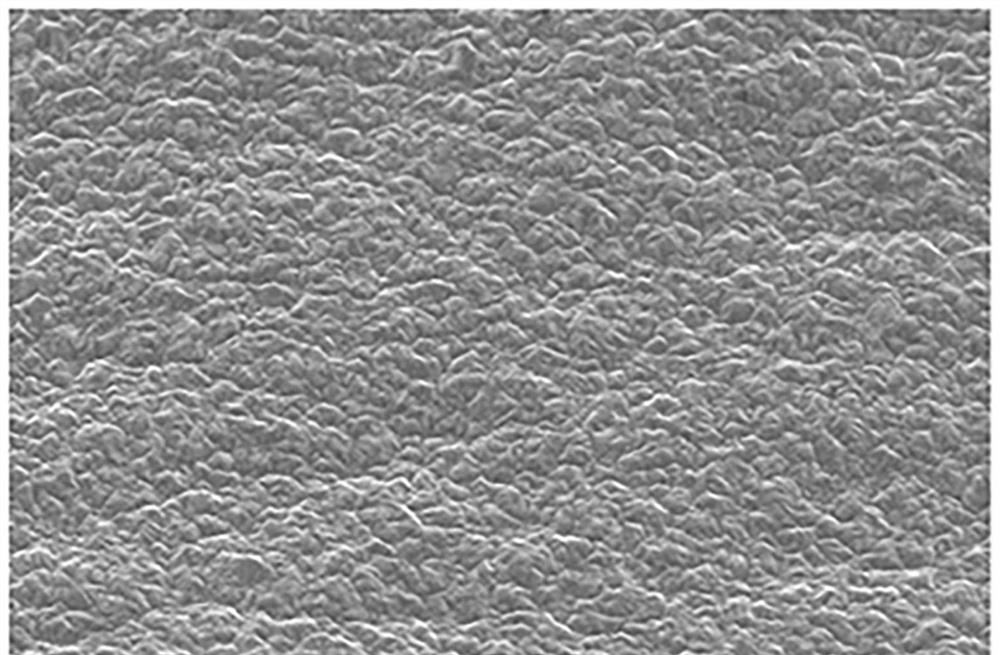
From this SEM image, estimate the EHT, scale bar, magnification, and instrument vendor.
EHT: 20 kV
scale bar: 10000 nm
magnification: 1 K X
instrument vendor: JEOL